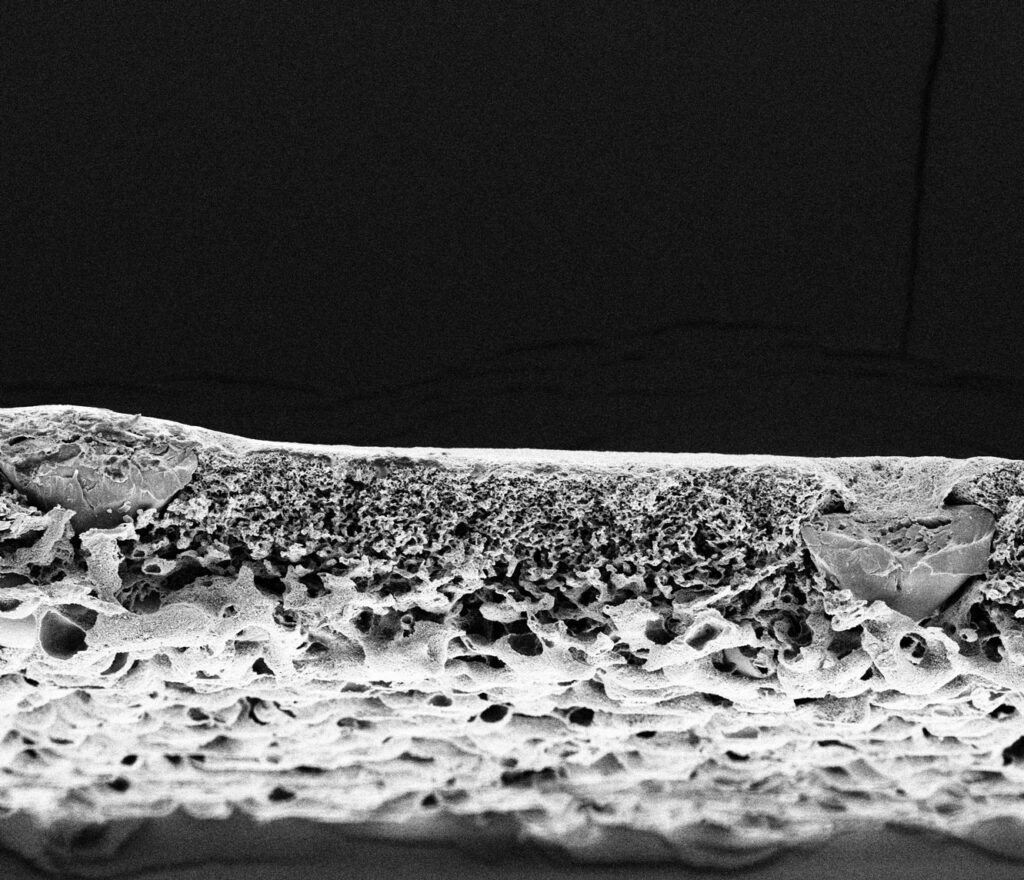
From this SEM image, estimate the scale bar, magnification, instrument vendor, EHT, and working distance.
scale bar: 50000 nm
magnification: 0.5 K X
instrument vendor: FEI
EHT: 5 kV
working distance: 6.1 mm